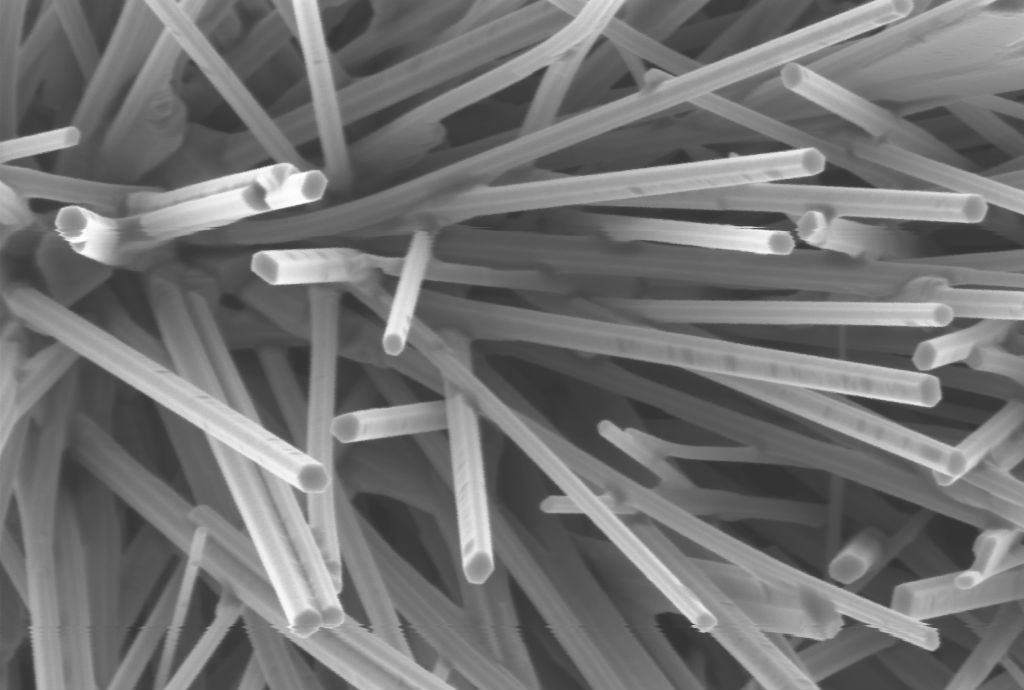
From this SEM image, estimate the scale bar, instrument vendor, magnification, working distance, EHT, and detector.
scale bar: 1000 nm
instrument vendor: Zeiss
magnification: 17.68 K X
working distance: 4.9 mm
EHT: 5 kV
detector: InLens